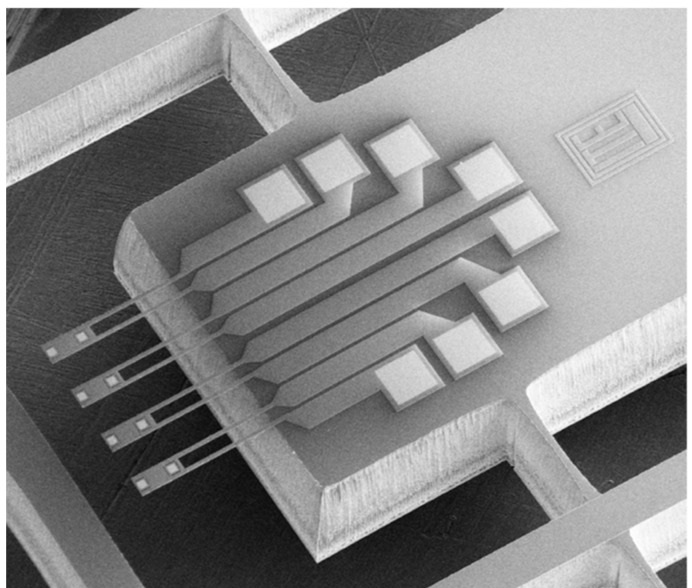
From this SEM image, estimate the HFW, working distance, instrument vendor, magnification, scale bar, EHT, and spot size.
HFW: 2910 µm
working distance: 5.7 mm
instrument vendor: FEI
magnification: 0.103 K X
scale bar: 1000000 nm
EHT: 10 kV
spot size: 3